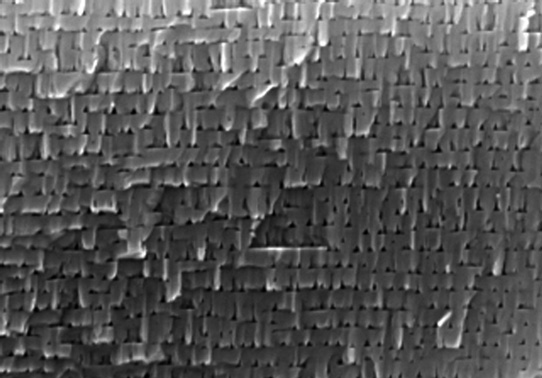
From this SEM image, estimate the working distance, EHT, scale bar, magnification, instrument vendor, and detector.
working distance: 6.9 mm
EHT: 15 kV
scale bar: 400 nm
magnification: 120 K X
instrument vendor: Hitachi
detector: SE(M)